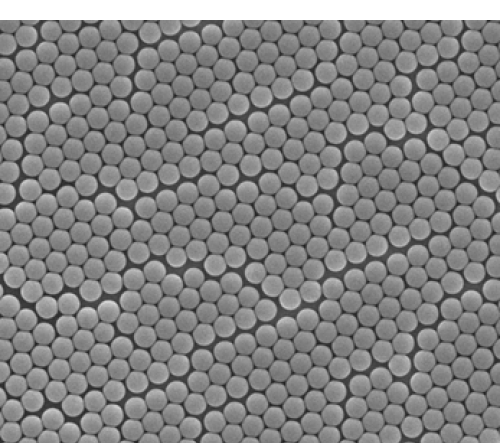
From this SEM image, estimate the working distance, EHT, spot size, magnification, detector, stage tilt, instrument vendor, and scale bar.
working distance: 8.7 mm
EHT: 10 kV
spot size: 3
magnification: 50 K X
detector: ETD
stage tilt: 0°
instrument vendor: FEI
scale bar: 2000 nm